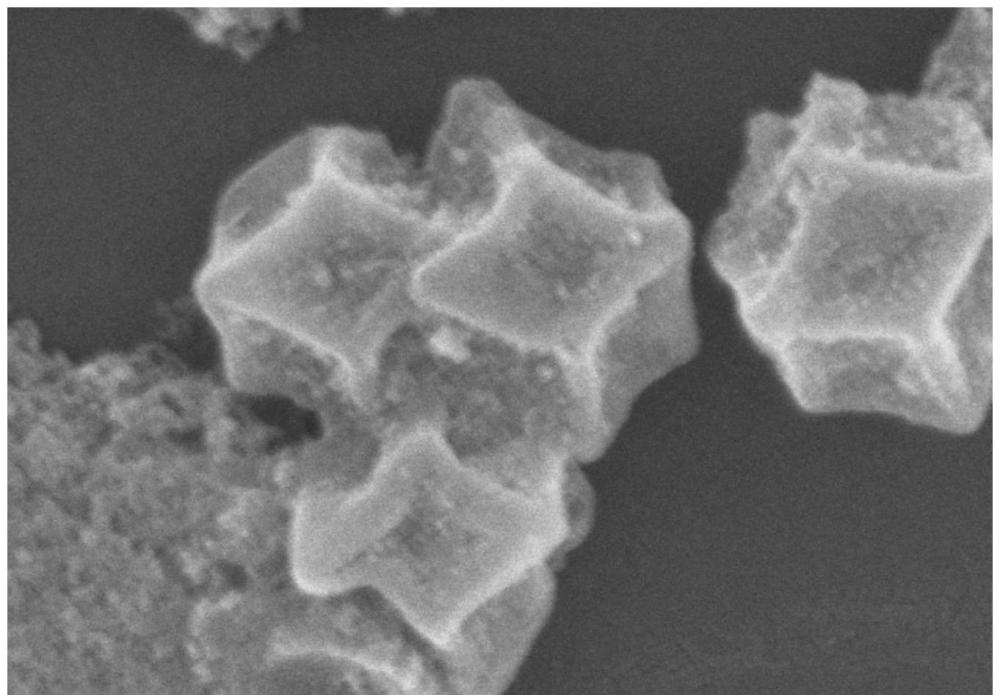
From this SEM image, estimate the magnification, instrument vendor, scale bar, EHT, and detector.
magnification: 80 K X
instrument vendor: Hitachi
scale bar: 500 nm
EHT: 12 kV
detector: SE(M)